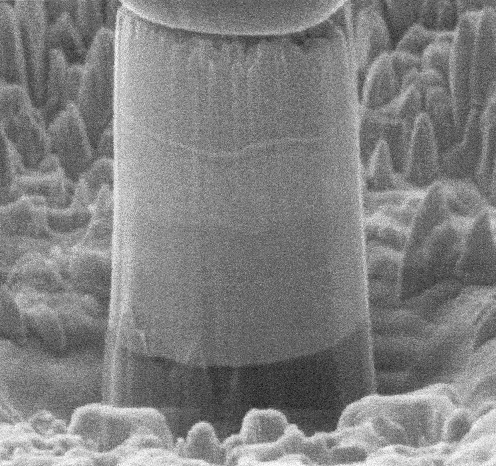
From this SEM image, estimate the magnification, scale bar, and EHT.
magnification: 10 K X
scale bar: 2000 nm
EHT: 5 kV